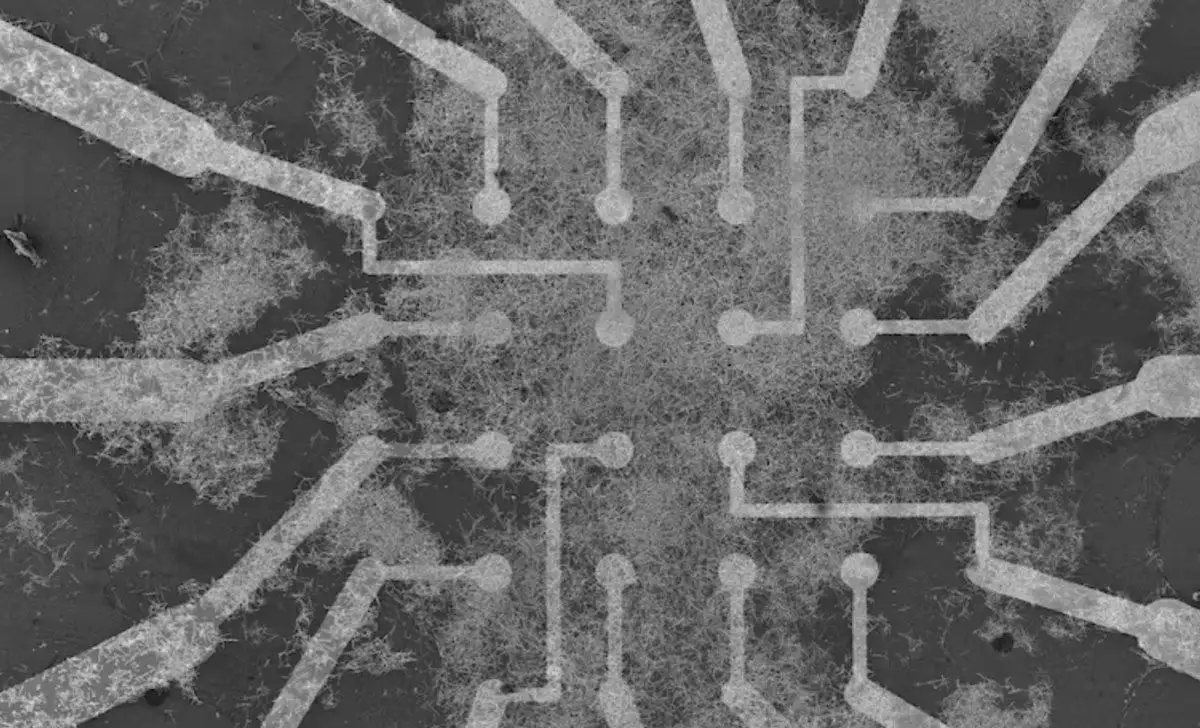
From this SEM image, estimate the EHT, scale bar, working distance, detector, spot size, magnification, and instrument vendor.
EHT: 10 kV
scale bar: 200000 nm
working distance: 14 mm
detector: SEI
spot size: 50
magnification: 0.065 K X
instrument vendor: JEOL